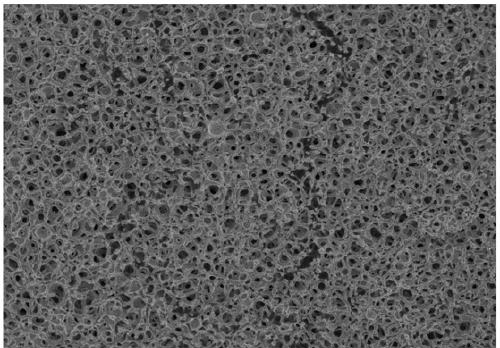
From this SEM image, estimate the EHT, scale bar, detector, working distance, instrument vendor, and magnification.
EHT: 3 kV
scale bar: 10000 nm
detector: SE(M)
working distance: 9.2 mm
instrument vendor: Hitachi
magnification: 5 K X